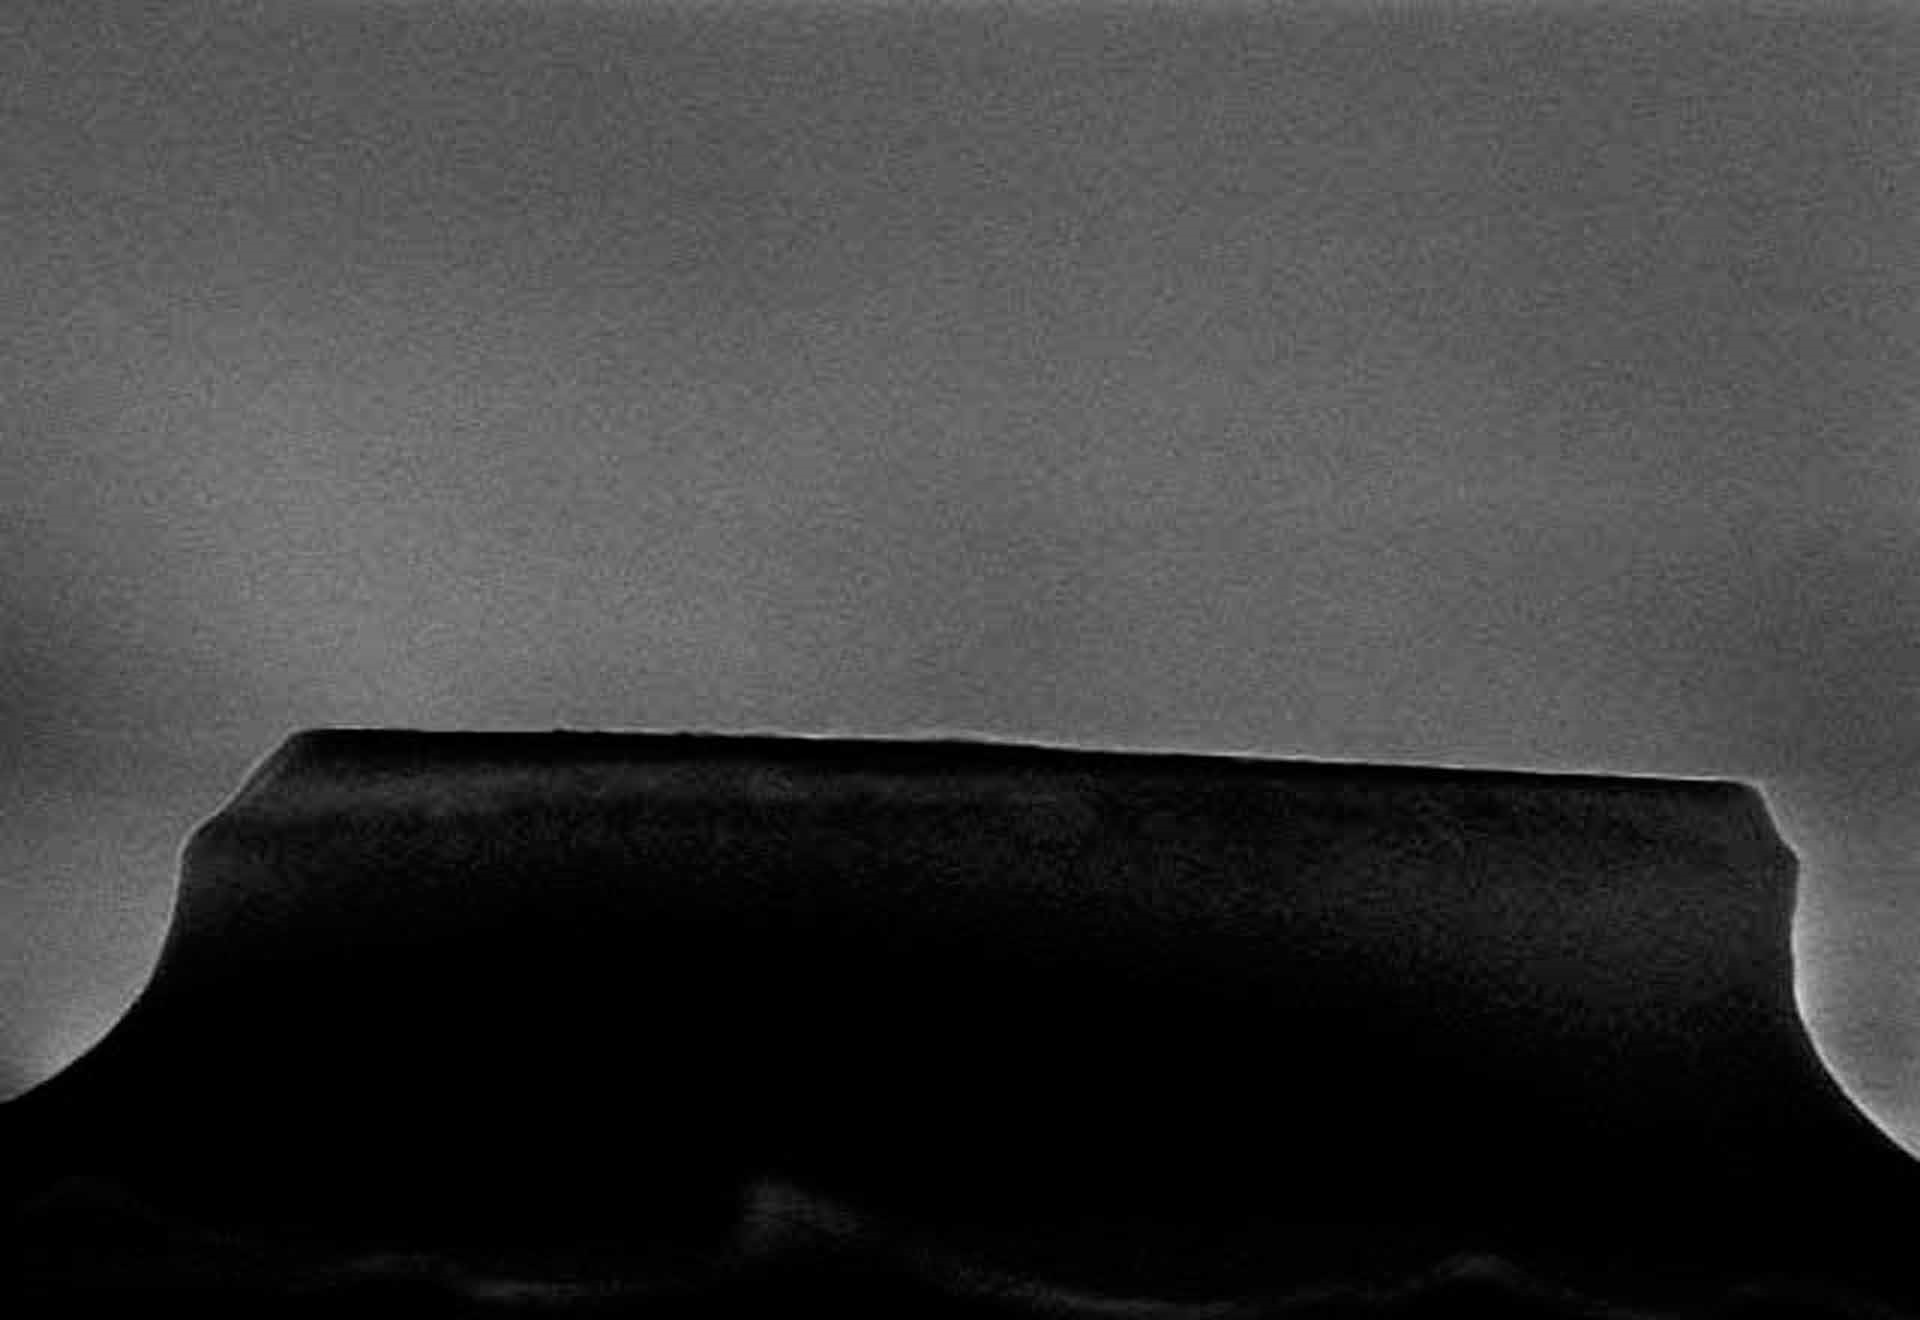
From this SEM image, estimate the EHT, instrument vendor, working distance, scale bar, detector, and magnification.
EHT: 15 kV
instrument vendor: Hitachi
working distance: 11.4 mm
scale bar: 4000 nm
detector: SE(M)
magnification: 13 K X